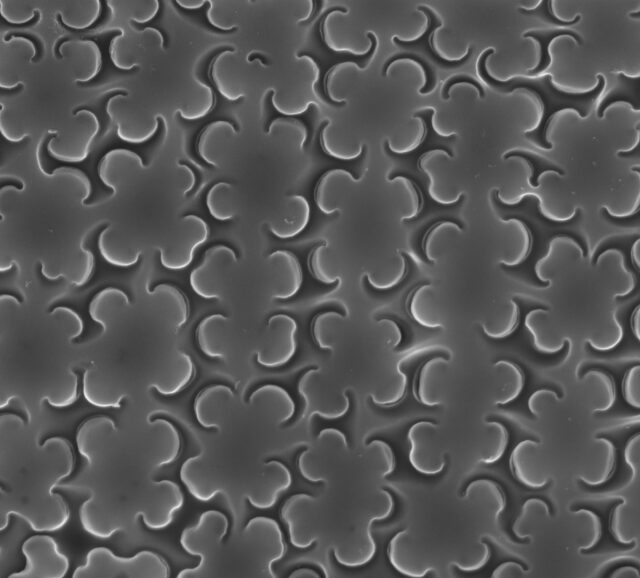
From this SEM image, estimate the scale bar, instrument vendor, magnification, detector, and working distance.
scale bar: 200 nm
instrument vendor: Zeiss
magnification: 20 K X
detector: InLens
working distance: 4 mm